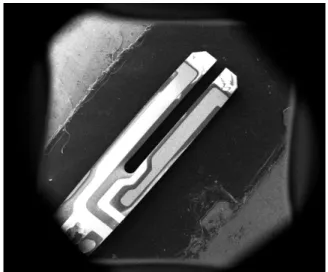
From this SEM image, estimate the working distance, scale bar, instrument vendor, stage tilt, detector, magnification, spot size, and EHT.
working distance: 13.9 mm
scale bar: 3000000 nm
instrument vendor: FEI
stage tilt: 0°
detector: ETD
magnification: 0.015 K X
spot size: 2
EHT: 10 kV